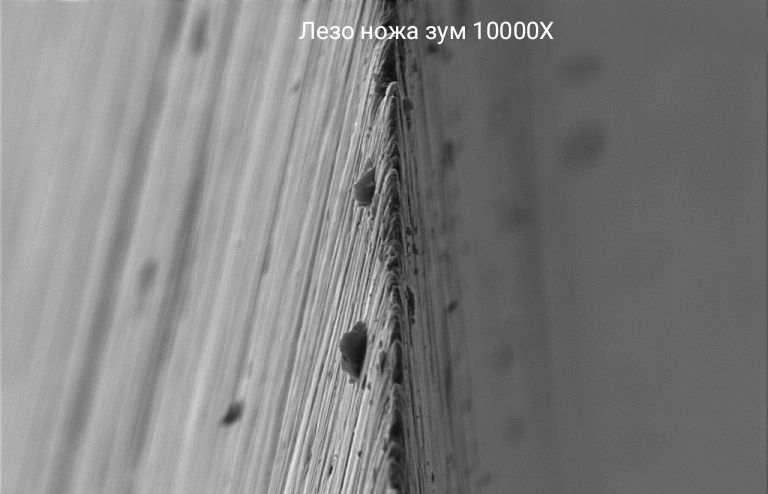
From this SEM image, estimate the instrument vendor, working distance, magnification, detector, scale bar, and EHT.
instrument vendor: Zeiss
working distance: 6.5 mm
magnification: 10 K X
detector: SE2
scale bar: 1000 nm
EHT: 5 kV